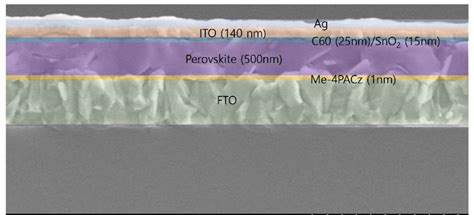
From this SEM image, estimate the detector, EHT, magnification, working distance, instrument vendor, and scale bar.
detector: SE(M)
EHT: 5 kV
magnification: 20 K X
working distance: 10.6 mm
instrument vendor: Hitachi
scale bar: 2000 nm